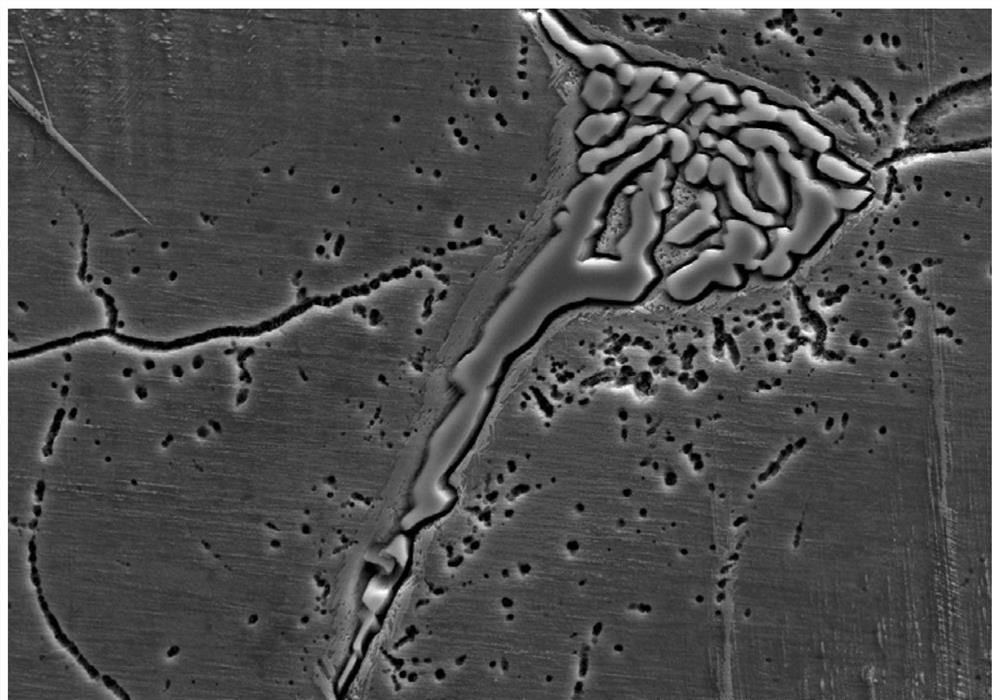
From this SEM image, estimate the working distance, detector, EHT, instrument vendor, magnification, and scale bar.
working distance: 10.6 mm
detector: SE(L)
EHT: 20 kV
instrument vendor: Hitachi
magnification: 2 K X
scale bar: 20000 nm